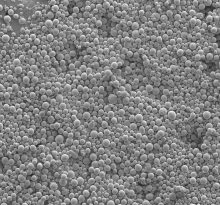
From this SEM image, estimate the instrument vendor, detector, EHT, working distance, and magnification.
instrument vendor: Hitachi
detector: SE(M)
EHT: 5 kV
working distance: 14.9 mm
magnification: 0.06 K X